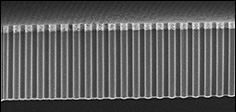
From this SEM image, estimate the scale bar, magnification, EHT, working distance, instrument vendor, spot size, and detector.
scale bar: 20000 nm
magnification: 1.026 K X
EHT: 5 kV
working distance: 4.9 mm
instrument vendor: FEI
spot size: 3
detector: TLD